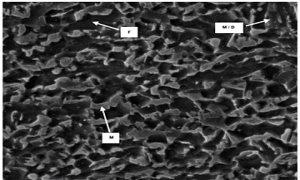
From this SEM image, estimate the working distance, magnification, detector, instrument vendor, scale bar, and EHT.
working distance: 11.8 mm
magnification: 5 K X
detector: ETD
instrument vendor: FEI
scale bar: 10000 nm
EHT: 20 kV